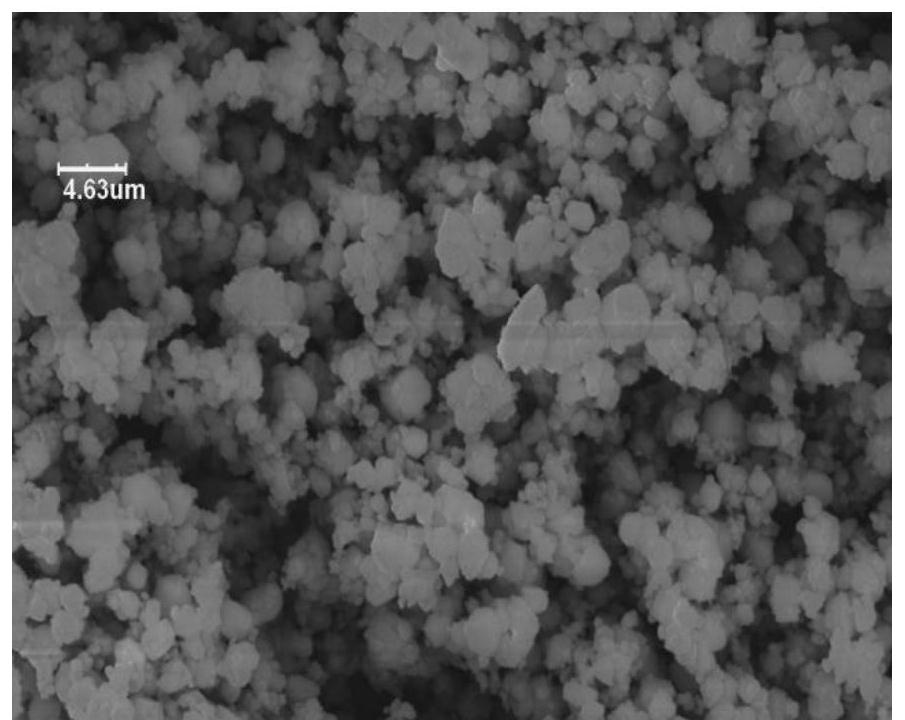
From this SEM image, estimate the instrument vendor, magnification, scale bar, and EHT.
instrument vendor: other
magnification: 2 K X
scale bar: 10000 nm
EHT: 19 kV